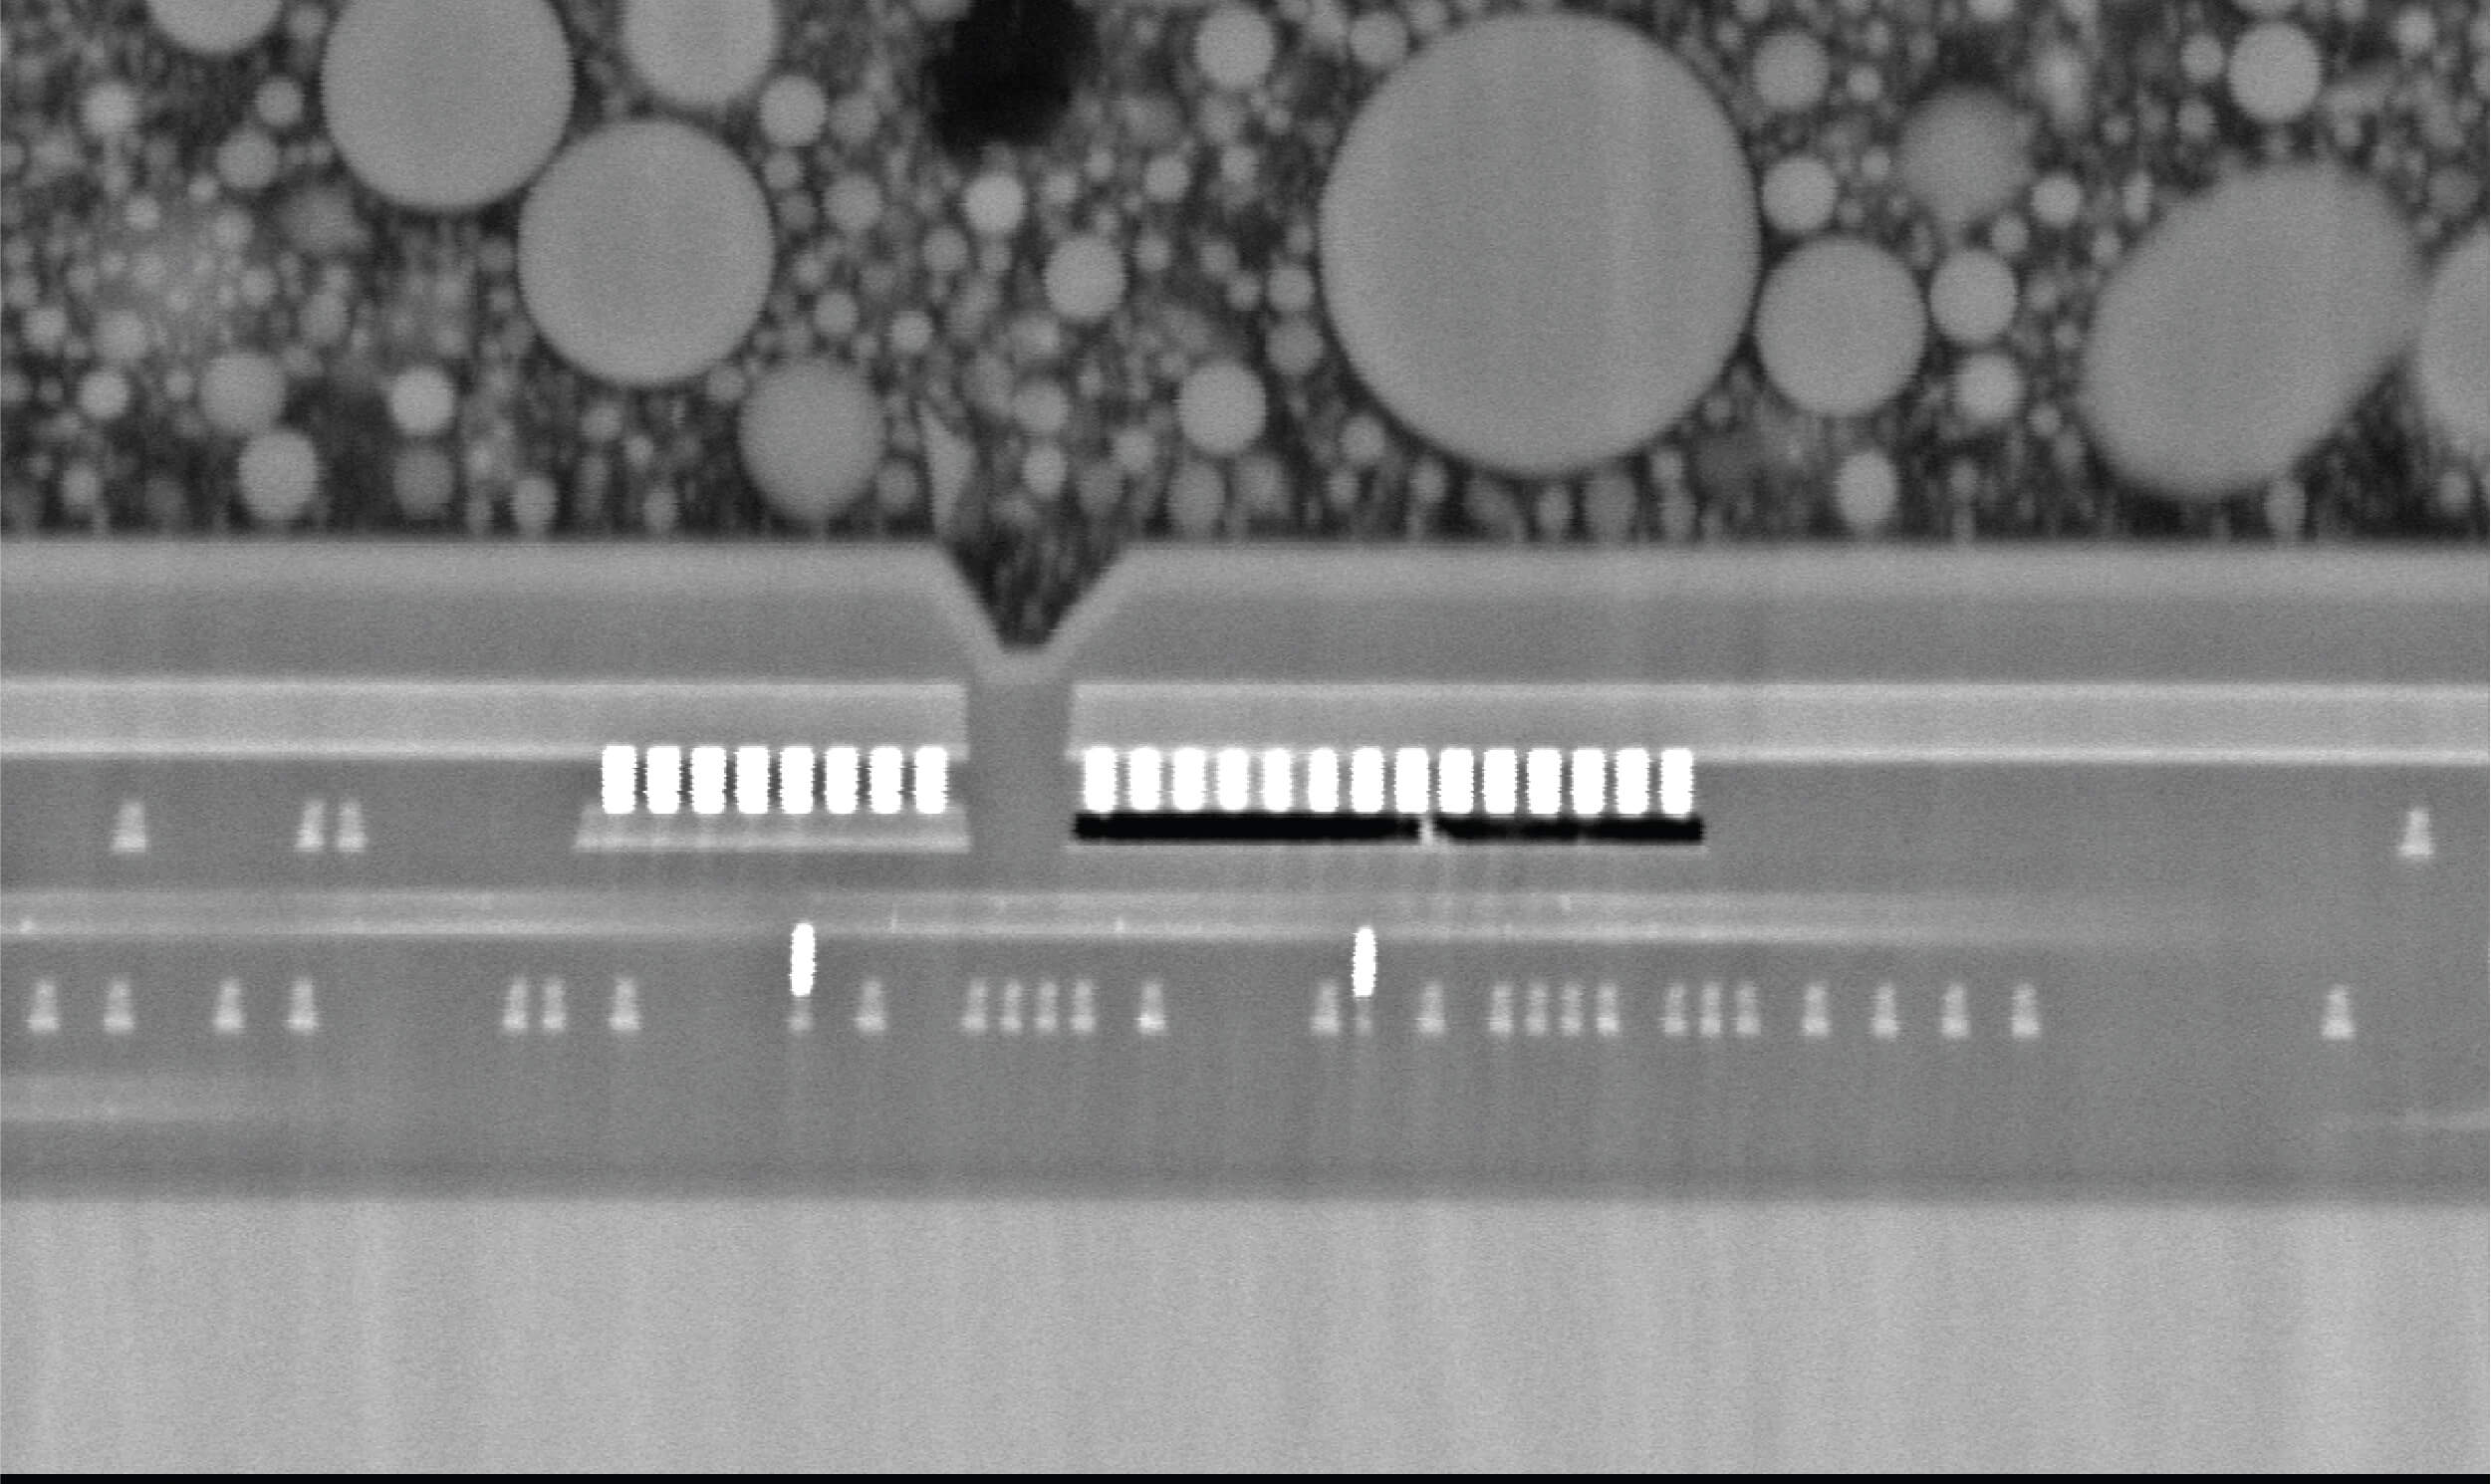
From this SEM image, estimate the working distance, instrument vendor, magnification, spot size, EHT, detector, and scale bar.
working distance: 9.2 mm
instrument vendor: other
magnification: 5 K X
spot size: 9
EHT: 15 kV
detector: BSE-COMPO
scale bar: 10000 nm